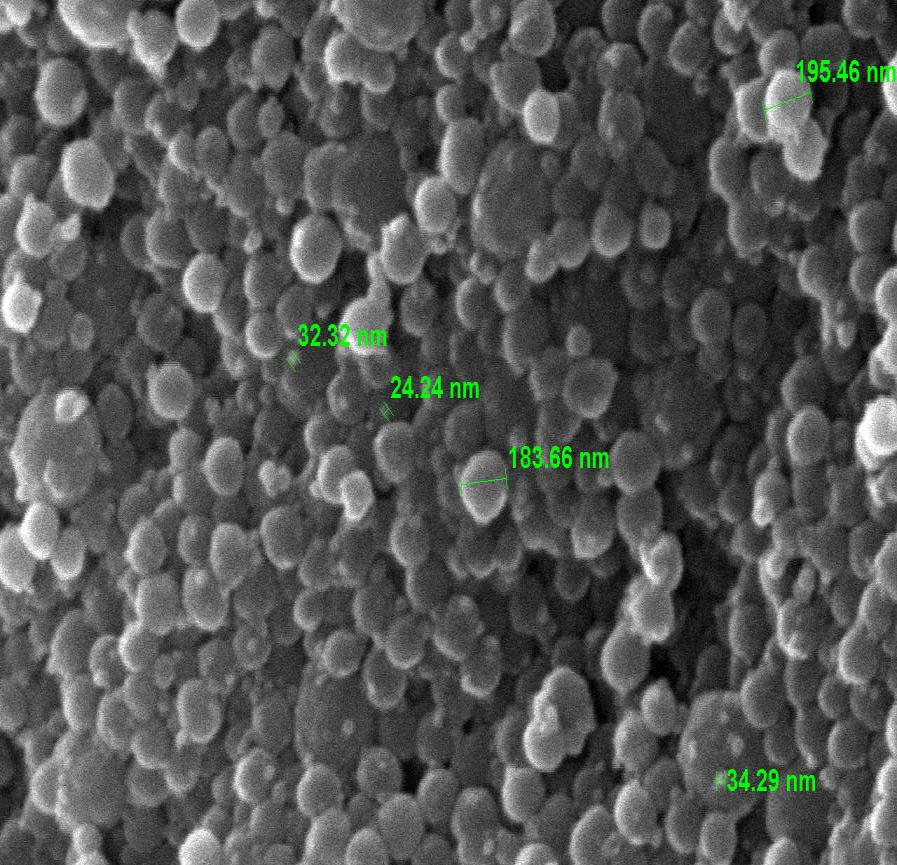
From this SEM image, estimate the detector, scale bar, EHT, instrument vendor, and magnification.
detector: SEI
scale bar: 500 nm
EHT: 15 kV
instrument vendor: JEOL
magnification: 35 K X